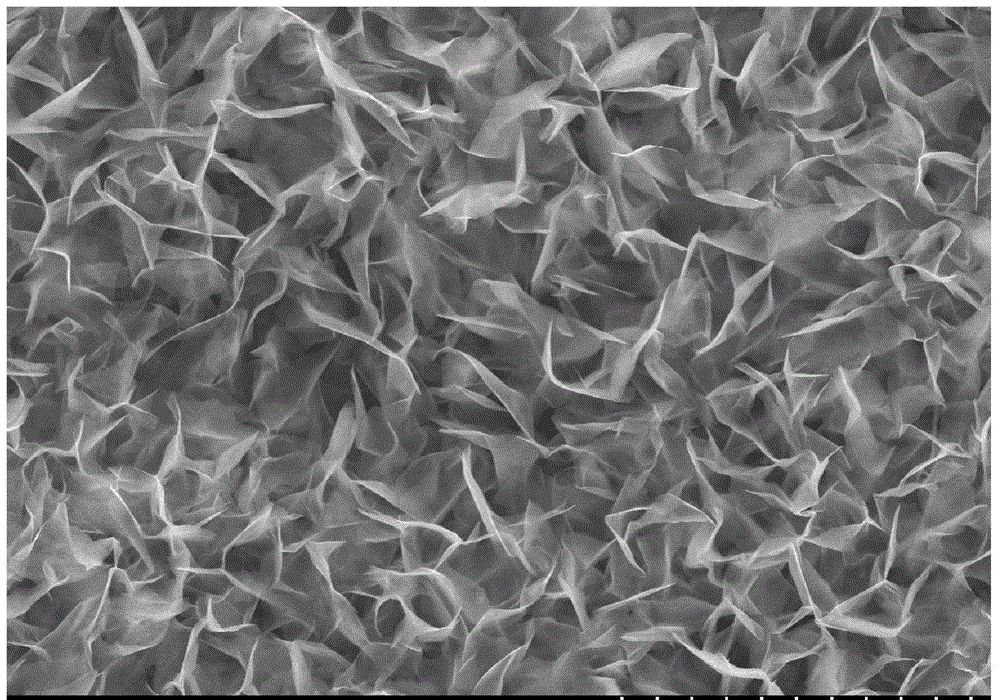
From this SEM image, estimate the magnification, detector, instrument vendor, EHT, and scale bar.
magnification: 4.5 K X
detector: SE(M,LA0)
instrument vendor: Hitachi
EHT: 15 kV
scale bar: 10000 nm